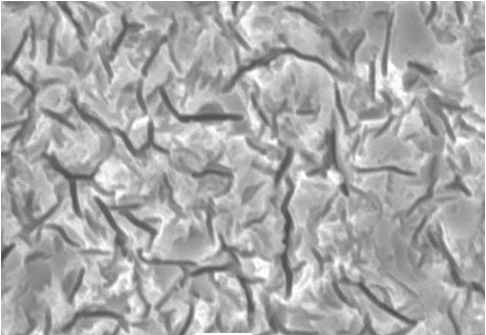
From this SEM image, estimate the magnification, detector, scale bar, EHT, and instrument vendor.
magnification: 3 K X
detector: SEI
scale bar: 5000 nm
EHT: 20 kV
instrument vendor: JEOL